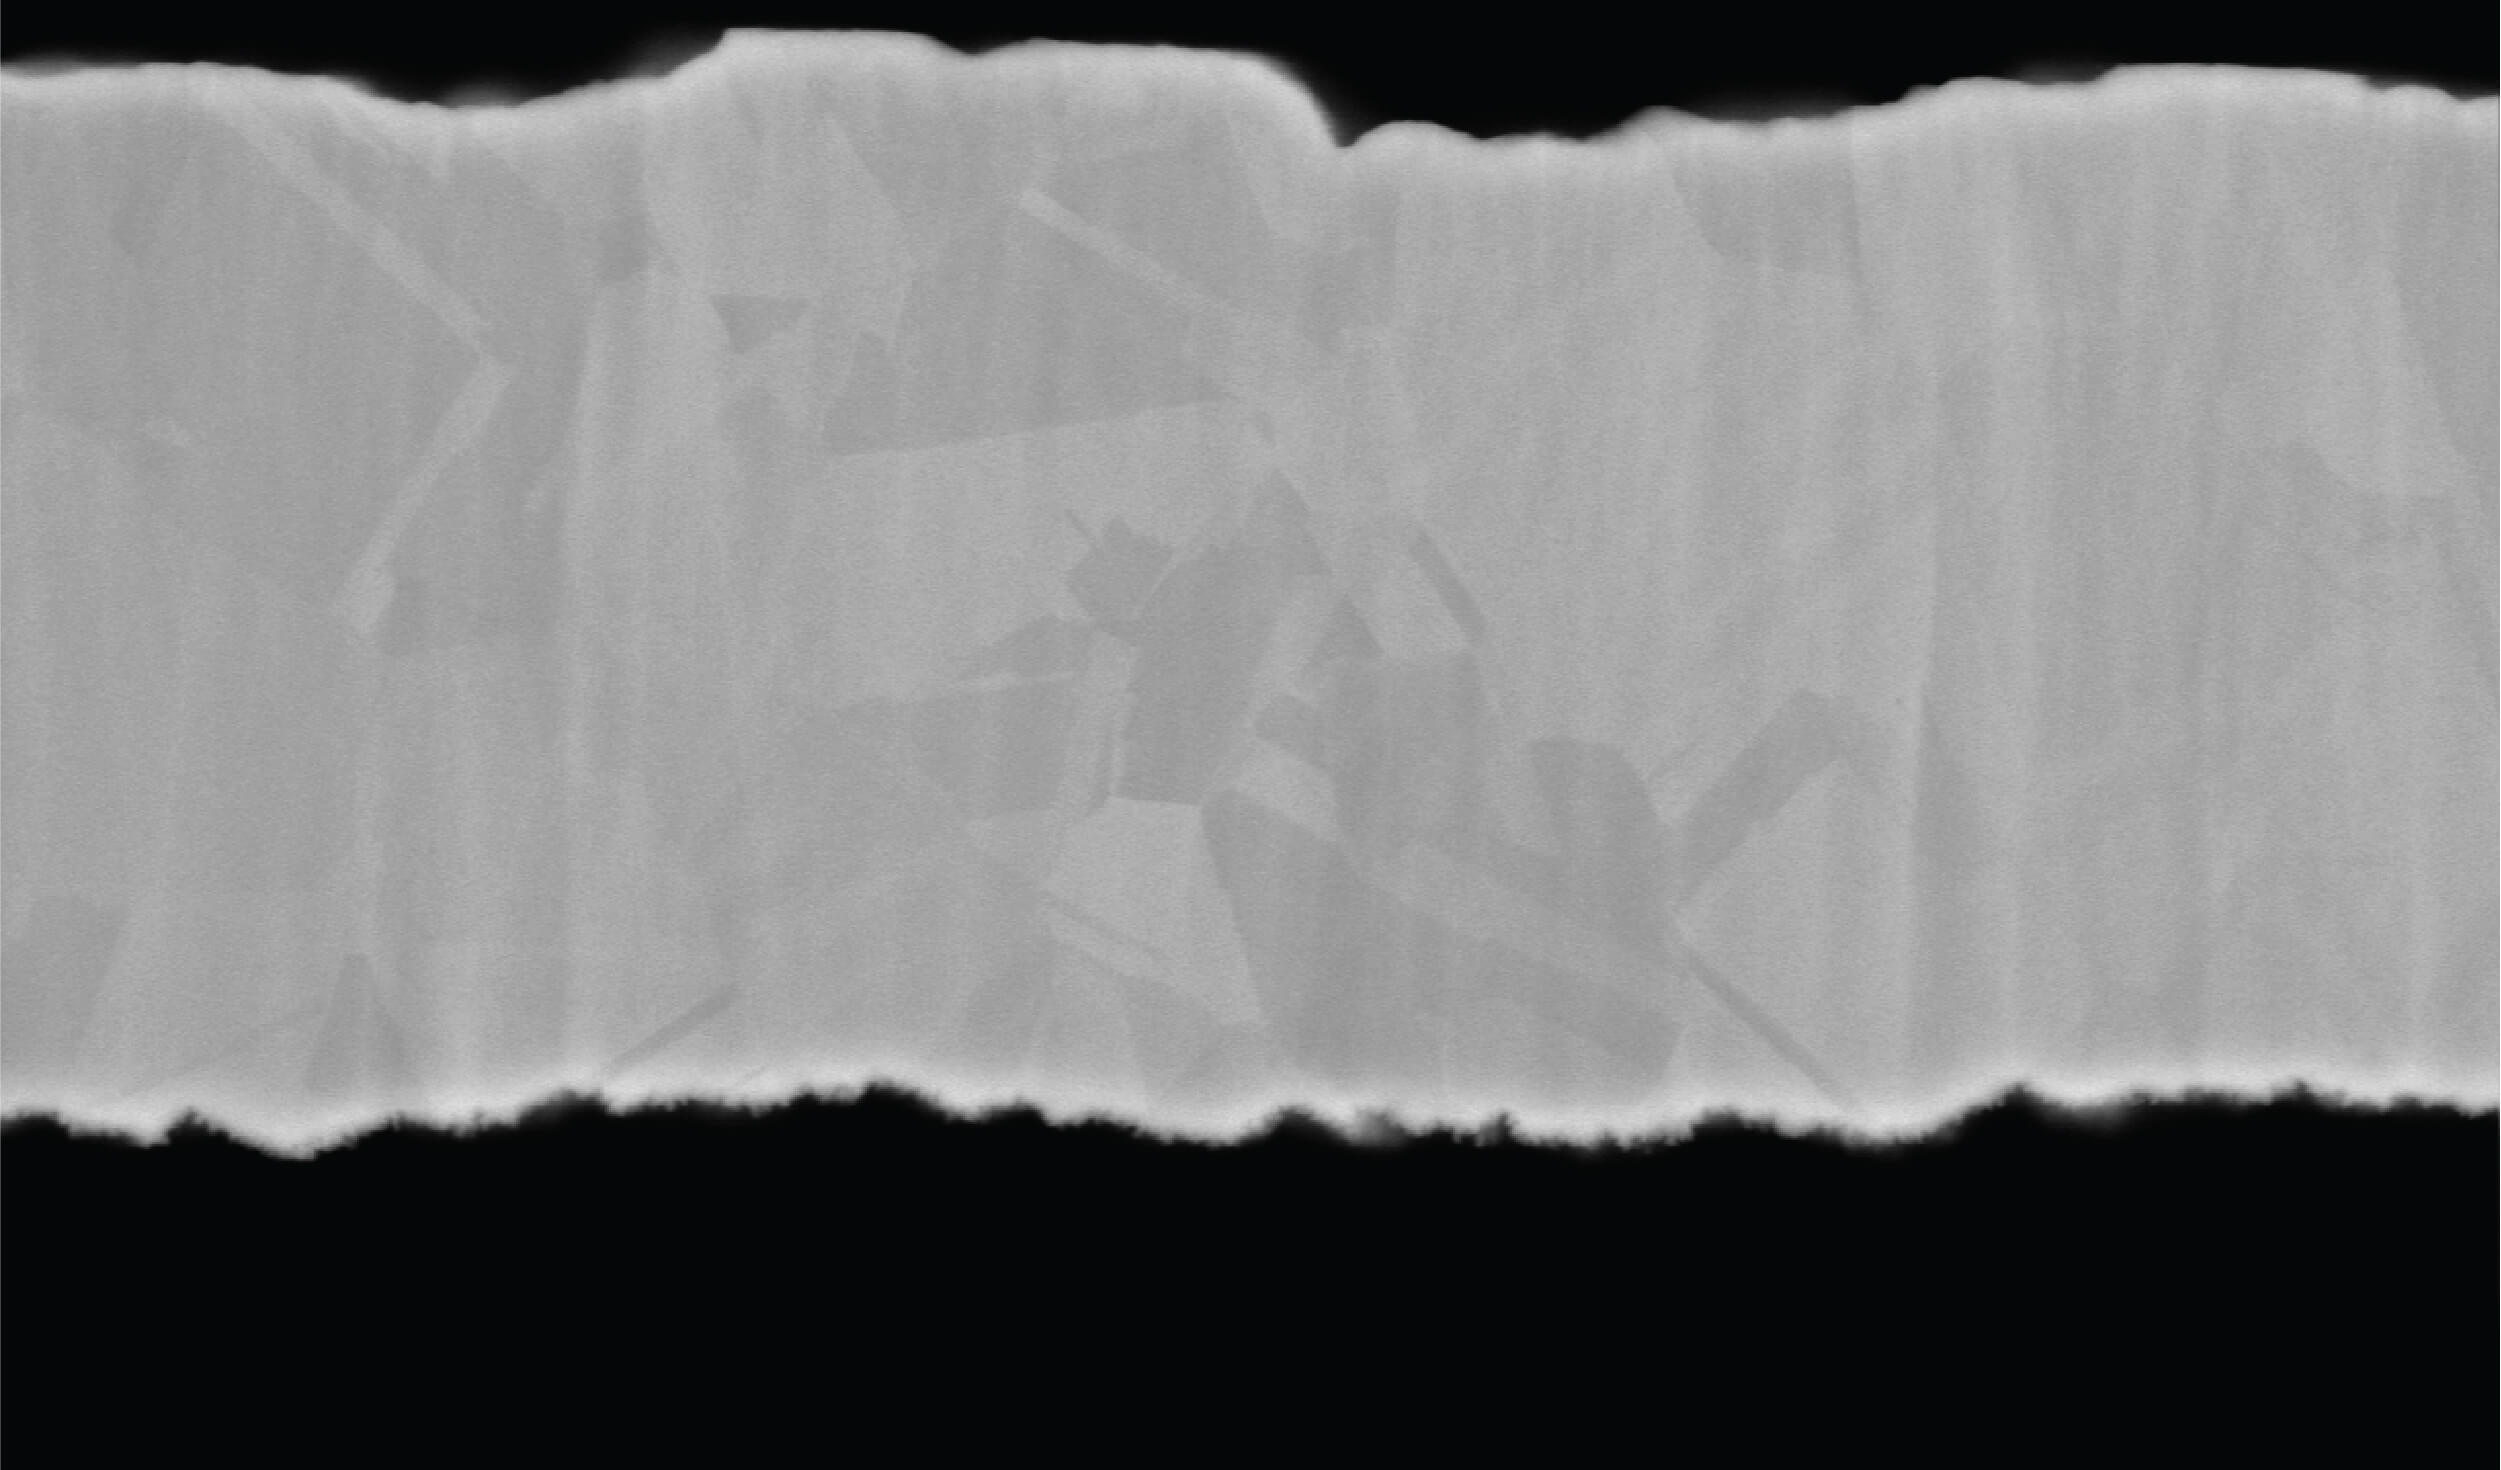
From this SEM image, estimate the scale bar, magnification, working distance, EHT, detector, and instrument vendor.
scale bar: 10000 nm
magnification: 6.6 K X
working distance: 7.6 mm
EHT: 20 kV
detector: BSE-COMPO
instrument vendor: other